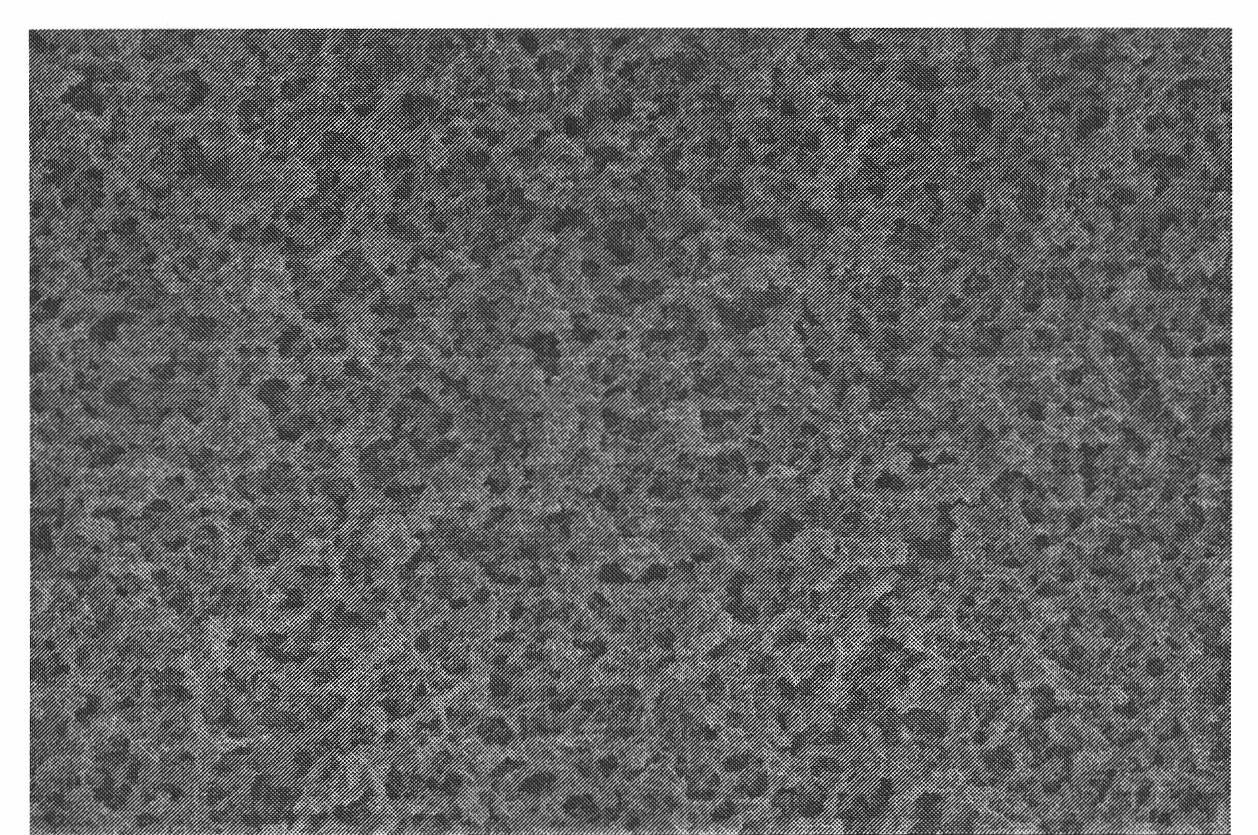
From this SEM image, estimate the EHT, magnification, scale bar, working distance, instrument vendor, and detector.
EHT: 5 kV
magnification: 1.5 K X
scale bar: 30000 nm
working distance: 10 mm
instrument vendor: Hitachi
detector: SE(M)